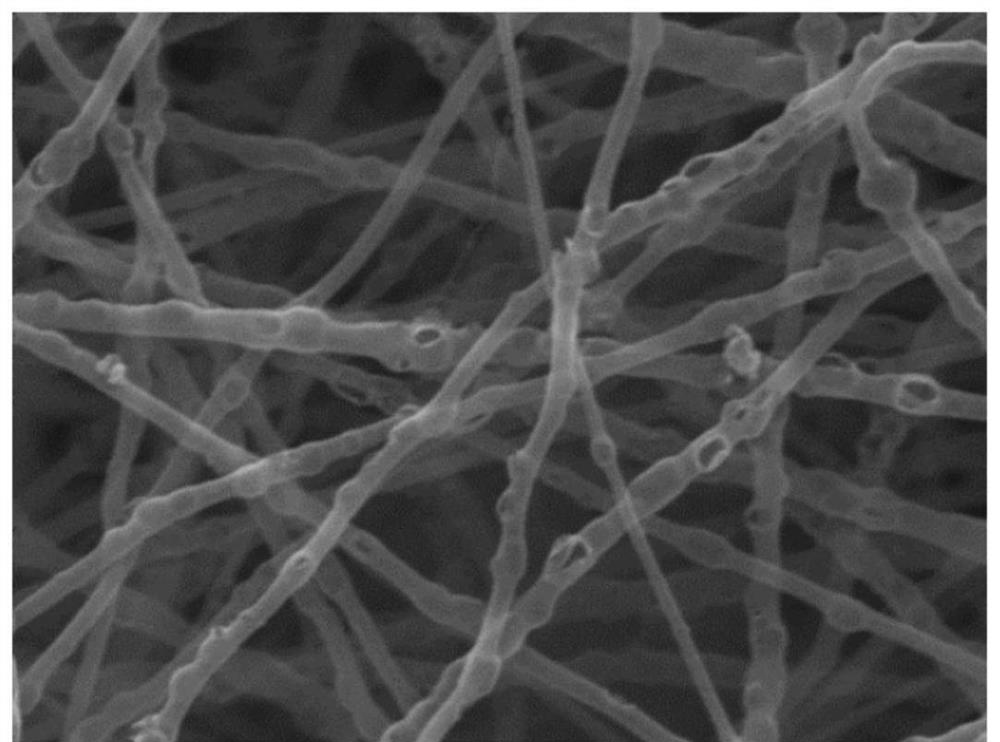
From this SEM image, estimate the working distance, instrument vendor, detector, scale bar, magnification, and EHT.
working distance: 6.01 mm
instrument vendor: Tescan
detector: SE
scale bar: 1000 nm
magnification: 50 K X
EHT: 20 kV